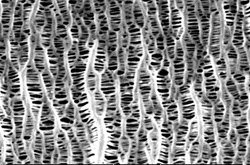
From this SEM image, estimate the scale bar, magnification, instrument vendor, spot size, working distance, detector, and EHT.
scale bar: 2000 nm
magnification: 10 K X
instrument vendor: FEI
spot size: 3.4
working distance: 21.3 mm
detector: SE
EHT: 20 kV